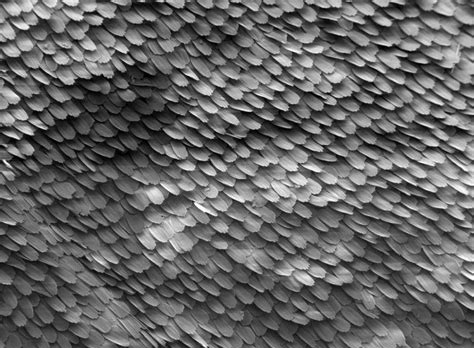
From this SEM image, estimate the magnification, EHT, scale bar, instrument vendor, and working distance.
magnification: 0.05 K X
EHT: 5 kV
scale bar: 500000 nm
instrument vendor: JEOL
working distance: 19 mm